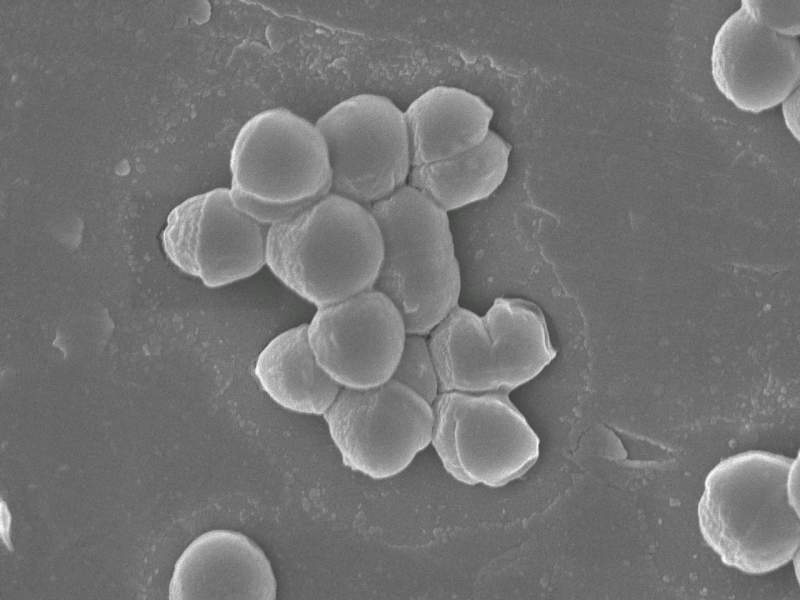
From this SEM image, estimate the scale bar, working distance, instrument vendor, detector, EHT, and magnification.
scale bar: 1000 nm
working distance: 3 mm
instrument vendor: JEOL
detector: SEI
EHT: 5 kV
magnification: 20 K X